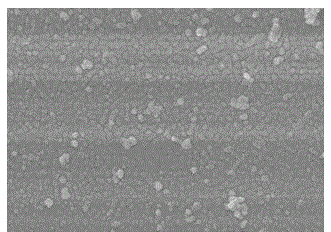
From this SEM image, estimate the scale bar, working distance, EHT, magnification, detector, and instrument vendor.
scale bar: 100 nm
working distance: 9.4 mm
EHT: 10 kV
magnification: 50 K X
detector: SEI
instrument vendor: JEOL